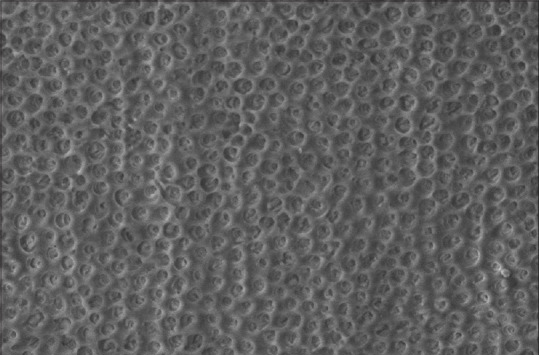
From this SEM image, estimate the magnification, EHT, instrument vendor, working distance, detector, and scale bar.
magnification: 1.5 K X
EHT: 15 kV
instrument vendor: Zeiss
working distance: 7 mm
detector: VPSE G3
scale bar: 10000 nm